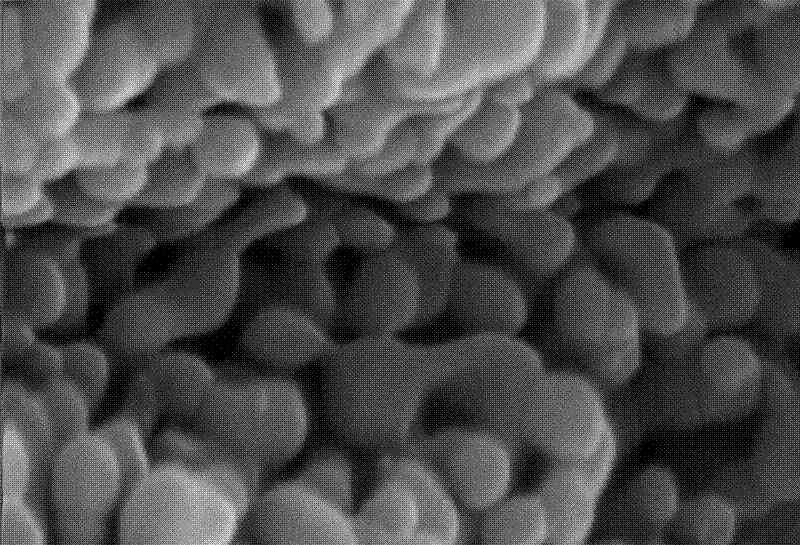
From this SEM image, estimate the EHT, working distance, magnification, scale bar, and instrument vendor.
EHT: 20 kV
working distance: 11.5 mm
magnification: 15 K X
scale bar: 2000 nm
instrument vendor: other